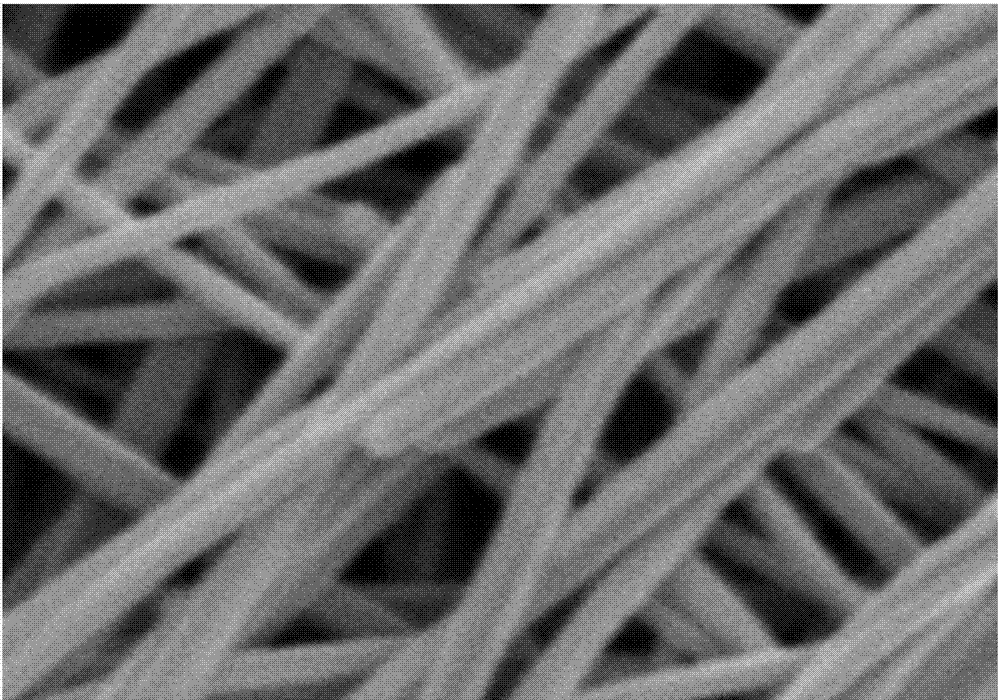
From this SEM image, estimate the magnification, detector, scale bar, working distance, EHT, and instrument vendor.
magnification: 80 K X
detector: SE(M)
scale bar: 500 nm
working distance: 9.5 mm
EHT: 3 kV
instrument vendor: Hitachi